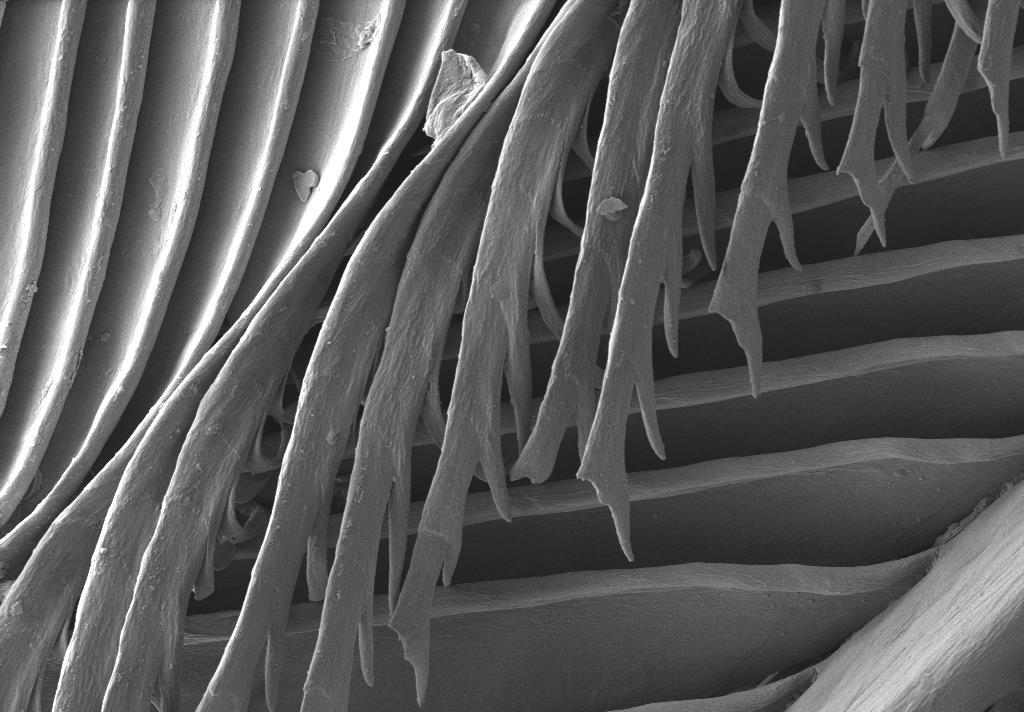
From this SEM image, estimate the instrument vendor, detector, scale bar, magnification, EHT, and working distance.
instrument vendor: Zeiss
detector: SE2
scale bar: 20000 nm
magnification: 0.482 K X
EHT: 5 kV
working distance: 5.8 mm